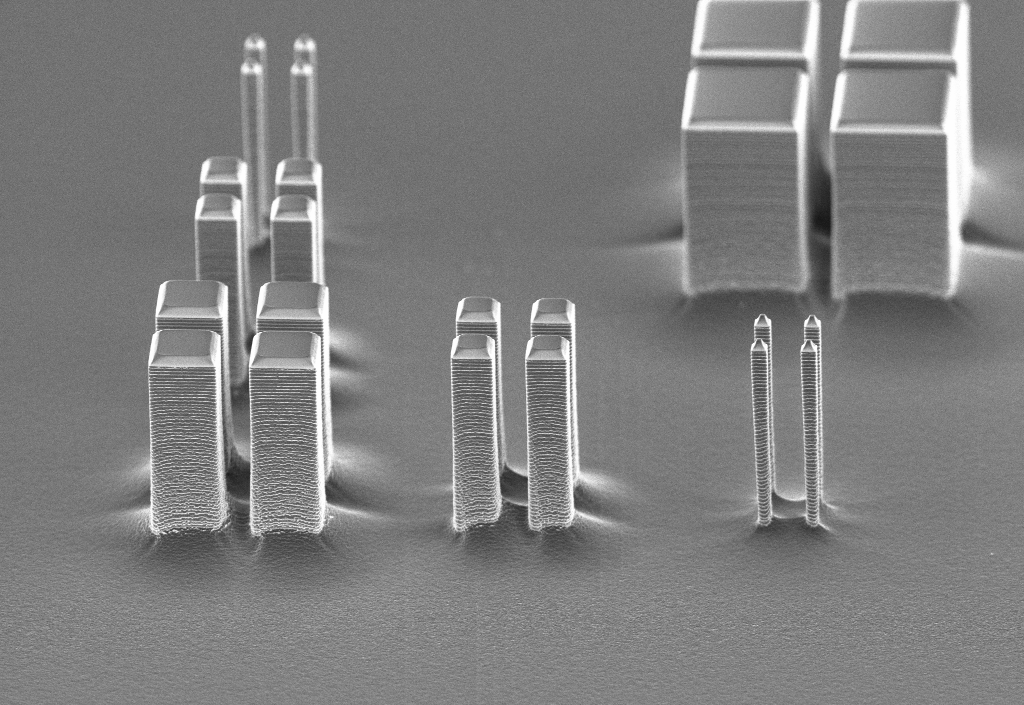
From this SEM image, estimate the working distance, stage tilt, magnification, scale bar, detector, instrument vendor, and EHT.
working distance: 1.7 mm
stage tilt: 30°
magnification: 3 K X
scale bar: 10000 nm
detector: InLens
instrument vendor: Zeiss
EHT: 3 kV